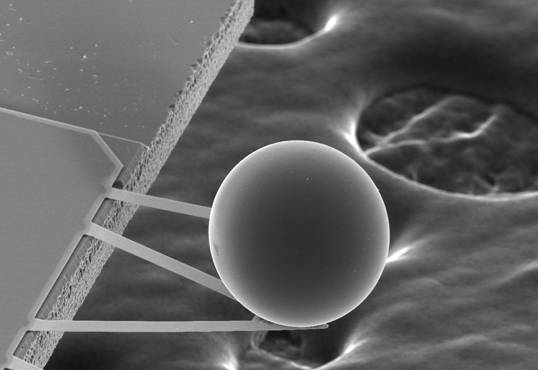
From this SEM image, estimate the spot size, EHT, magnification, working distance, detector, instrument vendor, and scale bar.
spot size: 3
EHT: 20 kV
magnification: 0.384 K X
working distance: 34.4 mm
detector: SE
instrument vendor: FEI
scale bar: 100000 nm